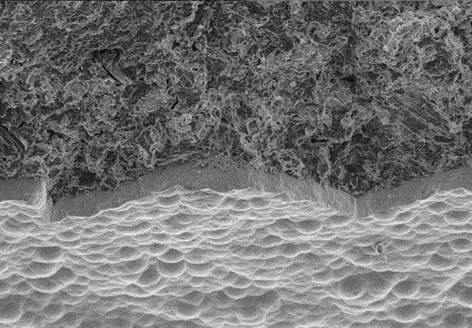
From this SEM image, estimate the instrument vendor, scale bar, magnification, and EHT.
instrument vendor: JEOL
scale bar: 40000 nm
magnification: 0.25 K X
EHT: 10 kV